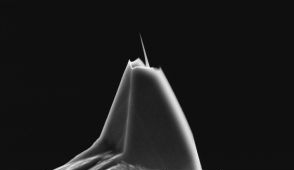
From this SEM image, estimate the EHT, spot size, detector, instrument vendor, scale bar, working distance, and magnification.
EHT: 10 kV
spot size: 3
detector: SE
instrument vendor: FEI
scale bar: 5000 nm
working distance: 22.4 mm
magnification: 5 K X